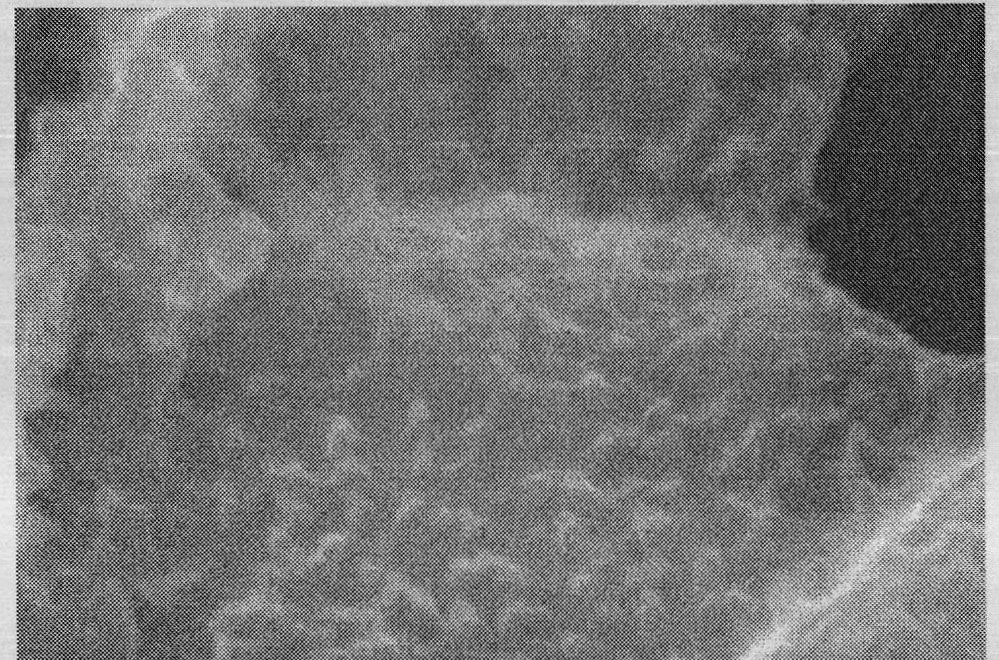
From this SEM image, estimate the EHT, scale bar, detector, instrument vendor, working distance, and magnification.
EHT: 15 kV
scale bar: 200 nm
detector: SE(M)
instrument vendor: Hitachi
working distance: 8.5 mm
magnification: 200 K X